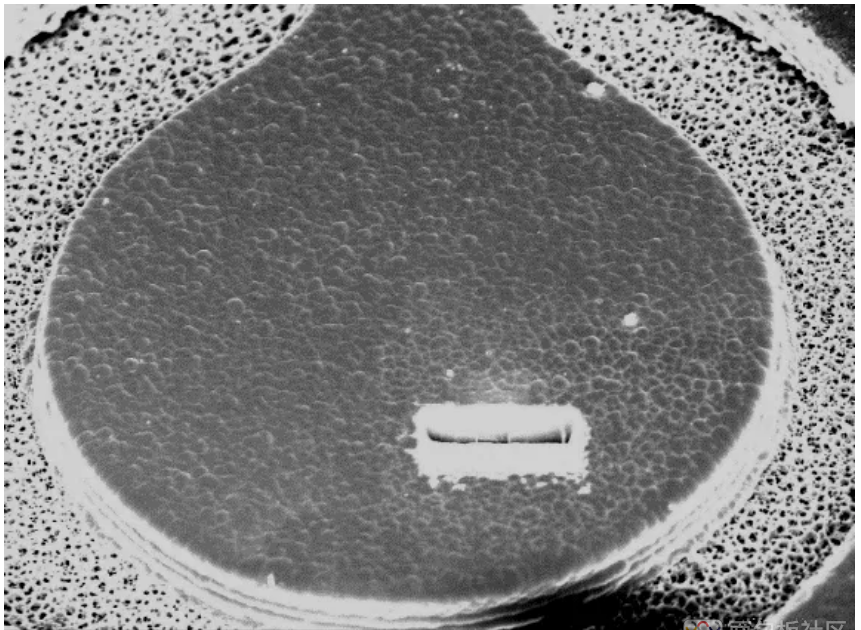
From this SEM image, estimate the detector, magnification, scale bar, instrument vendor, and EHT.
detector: SEI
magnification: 0.43 K X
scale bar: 10000 nm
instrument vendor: JEOL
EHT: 15 kV